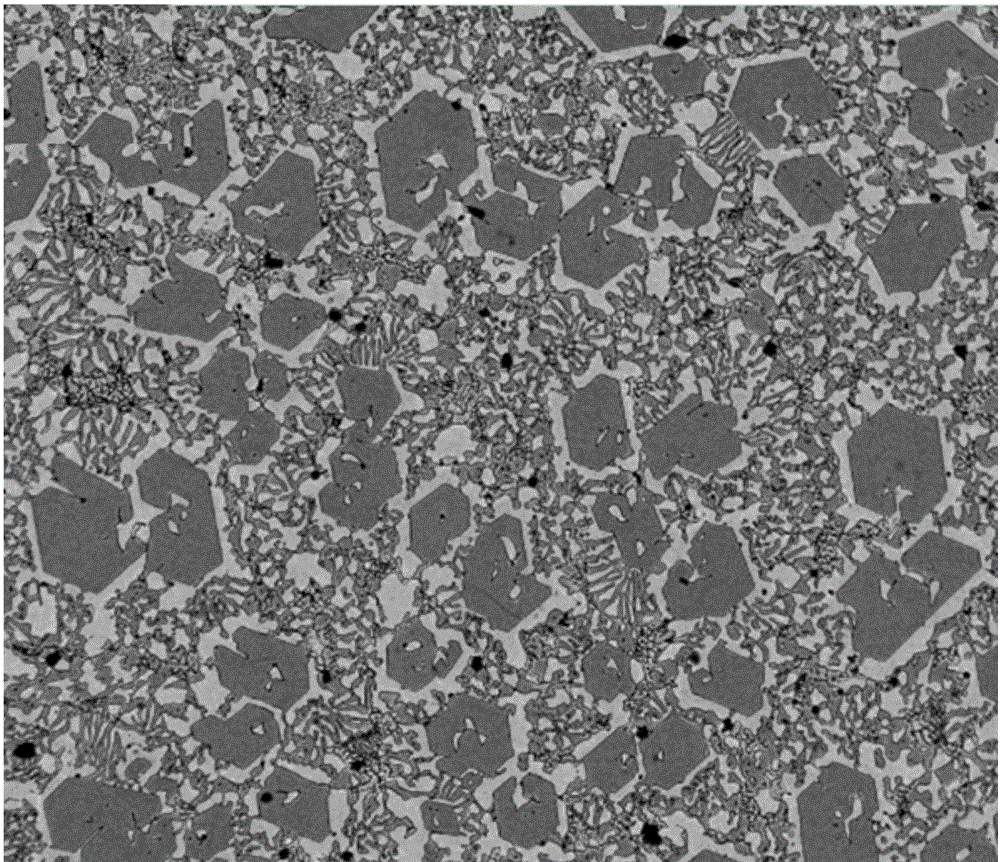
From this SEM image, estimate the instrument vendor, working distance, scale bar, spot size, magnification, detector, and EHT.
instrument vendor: FEI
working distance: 9.4 mm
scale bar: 50000 nm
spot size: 6.5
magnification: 0.5 K X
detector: SSD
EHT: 15 kV